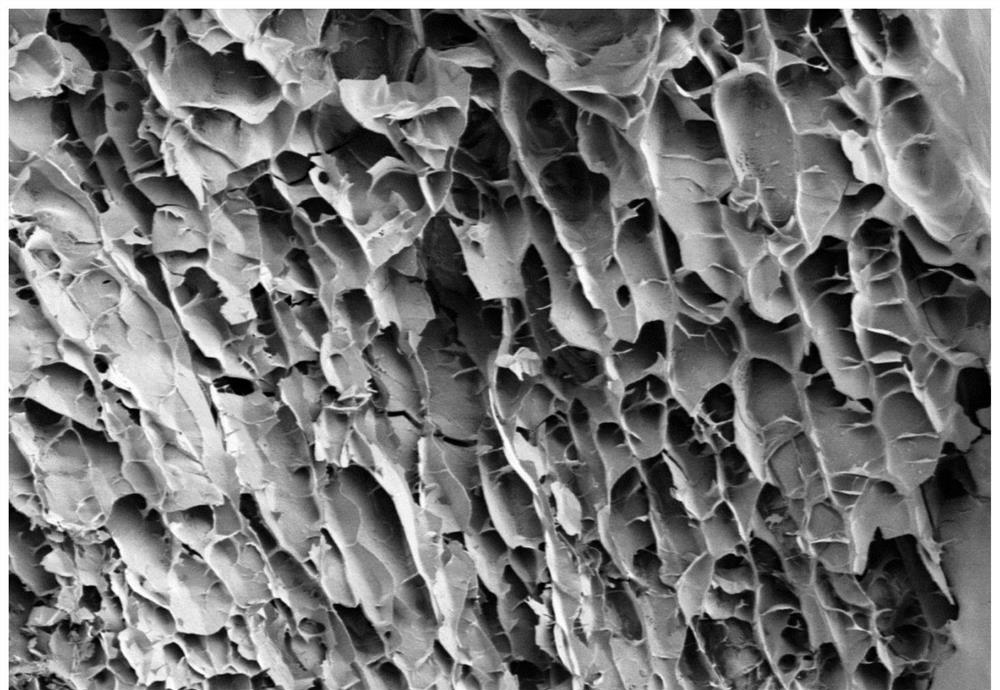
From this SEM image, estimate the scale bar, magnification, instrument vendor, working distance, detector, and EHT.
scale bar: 1000000 nm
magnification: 0.05 K X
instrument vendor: Hitachi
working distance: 13.3 mm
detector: LM(L)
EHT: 3 kV